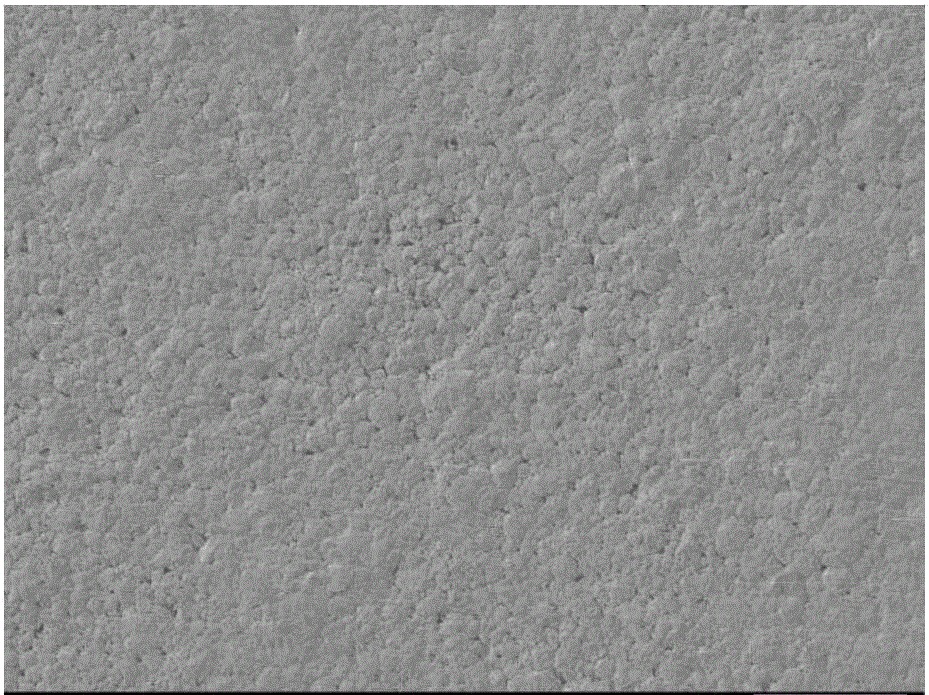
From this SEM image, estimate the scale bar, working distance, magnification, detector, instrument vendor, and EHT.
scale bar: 100000 nm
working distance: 8 mm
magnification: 0.2 K X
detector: LEI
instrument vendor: JEOL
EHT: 5 kV